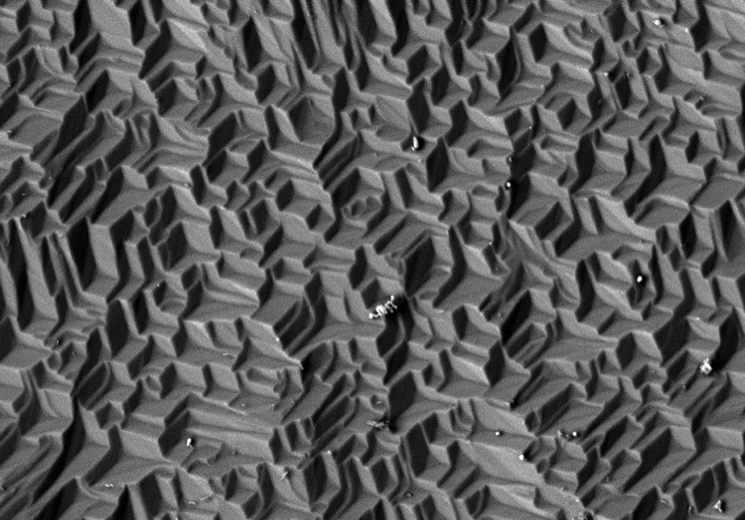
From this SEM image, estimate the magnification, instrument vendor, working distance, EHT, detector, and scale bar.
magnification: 1.2 K X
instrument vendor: Hitachi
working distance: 10.2 mm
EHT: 10 kV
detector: BSE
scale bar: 40000 nm